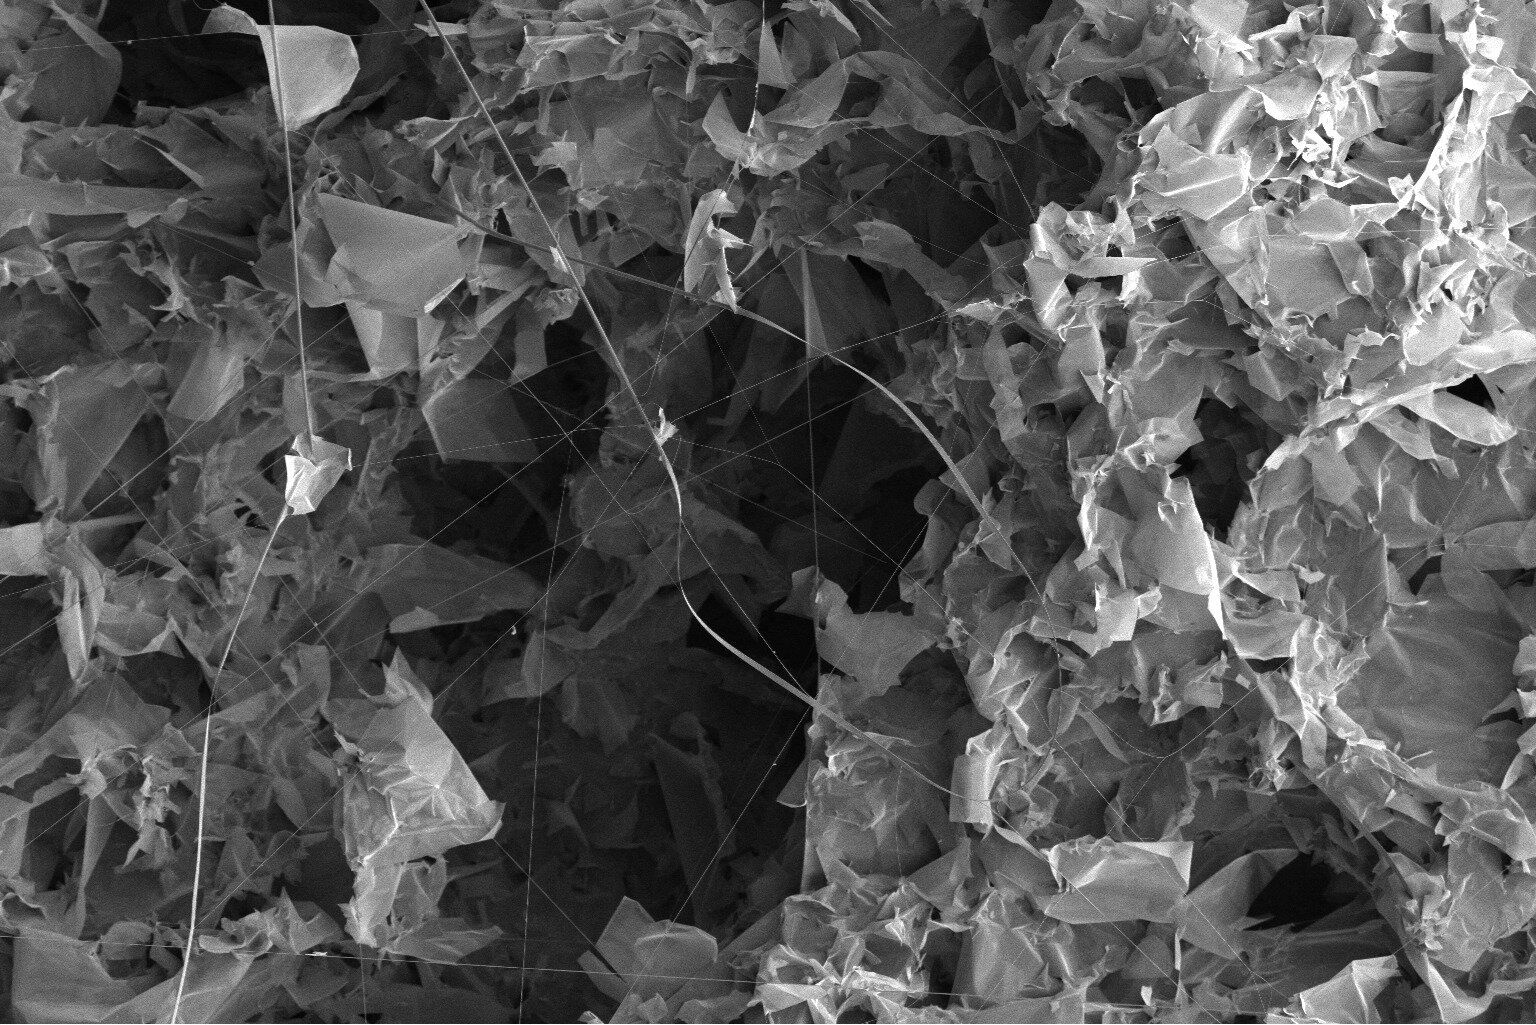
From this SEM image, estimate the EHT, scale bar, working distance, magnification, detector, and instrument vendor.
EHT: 2 kV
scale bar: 50000 nm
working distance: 5 mm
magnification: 0.8 K X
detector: ETD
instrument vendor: FEI